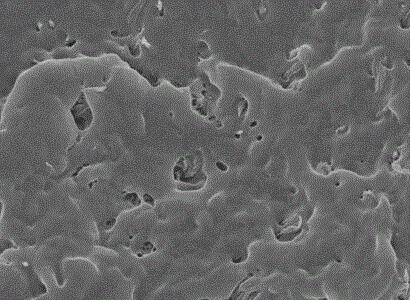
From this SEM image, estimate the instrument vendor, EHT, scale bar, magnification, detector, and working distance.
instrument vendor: Hitachi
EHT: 4 kV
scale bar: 2000 nm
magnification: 20 K X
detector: SE(U)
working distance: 8.9 mm